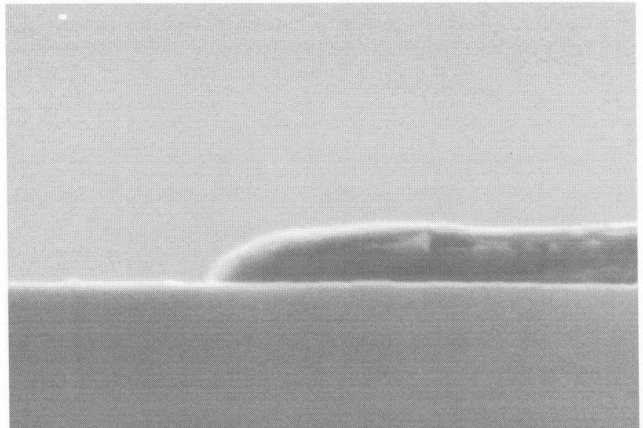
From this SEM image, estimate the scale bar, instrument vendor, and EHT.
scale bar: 300 nm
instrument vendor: JEOL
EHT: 20 kV